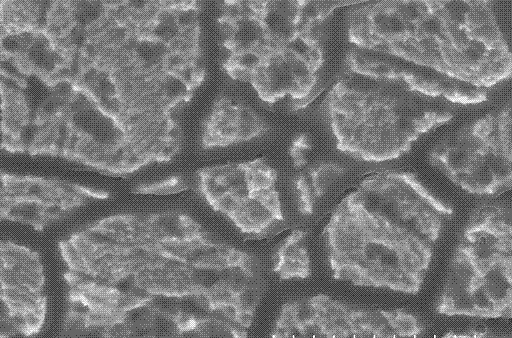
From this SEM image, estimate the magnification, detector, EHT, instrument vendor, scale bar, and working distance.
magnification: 5 K X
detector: SE(U)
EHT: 15 kV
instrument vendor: Hitachi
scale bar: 10000 nm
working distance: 9.6 mm